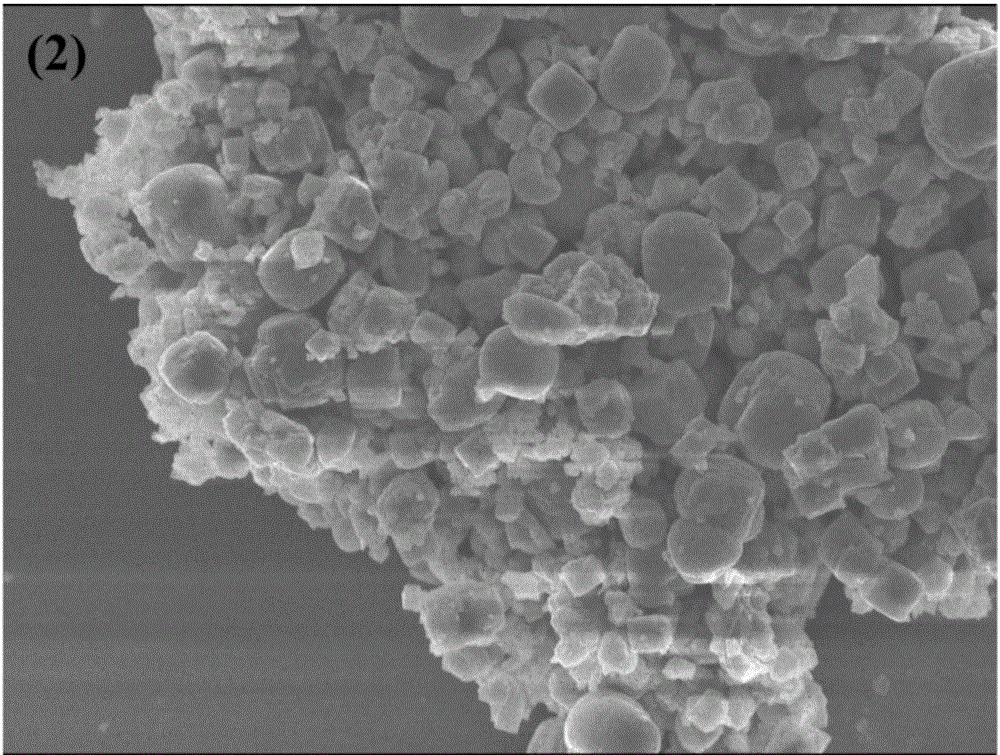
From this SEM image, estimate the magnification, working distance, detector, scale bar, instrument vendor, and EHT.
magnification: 8 K X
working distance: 7.6 mm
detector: SEI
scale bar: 1000 nm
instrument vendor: JEOL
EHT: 8 kV